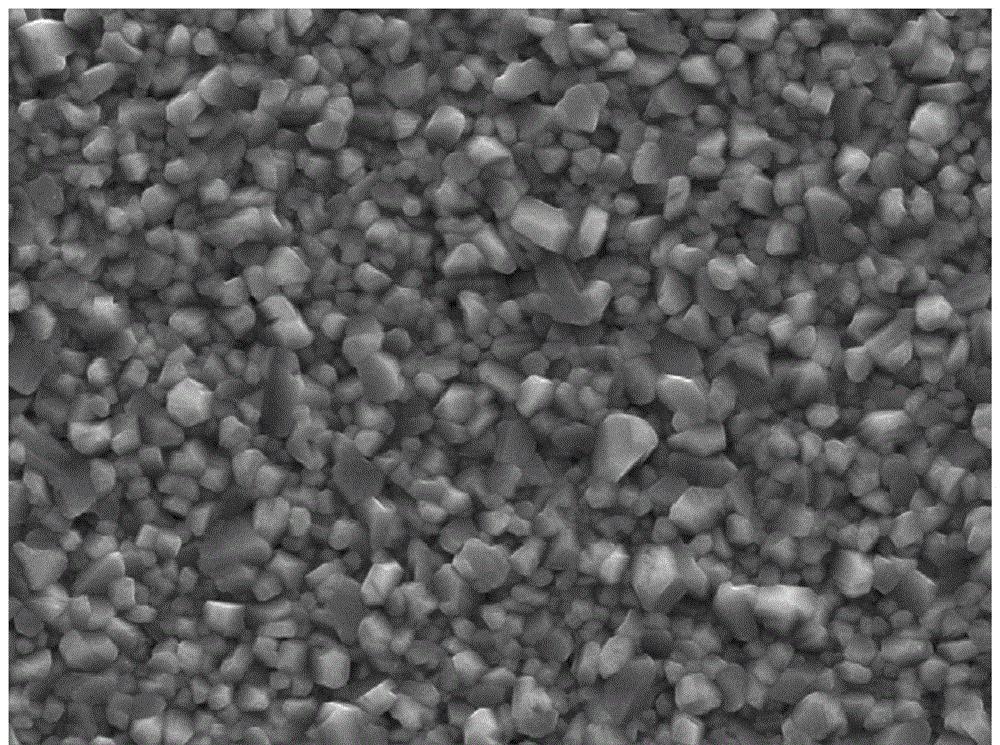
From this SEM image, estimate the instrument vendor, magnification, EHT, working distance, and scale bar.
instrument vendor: Tescan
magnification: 10 K X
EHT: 20 kV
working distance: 14.88 mm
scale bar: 5000 nm